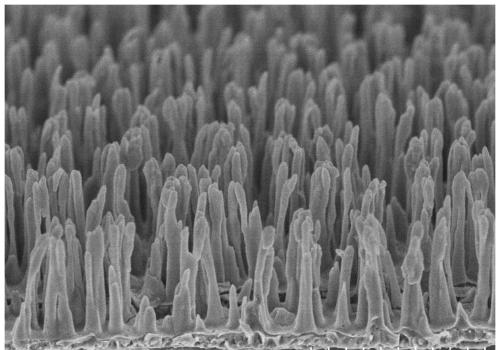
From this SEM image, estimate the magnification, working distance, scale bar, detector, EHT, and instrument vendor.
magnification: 15 K X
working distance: -0.1 mm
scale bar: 3000 nm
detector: SE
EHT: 3 kV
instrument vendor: Hitachi